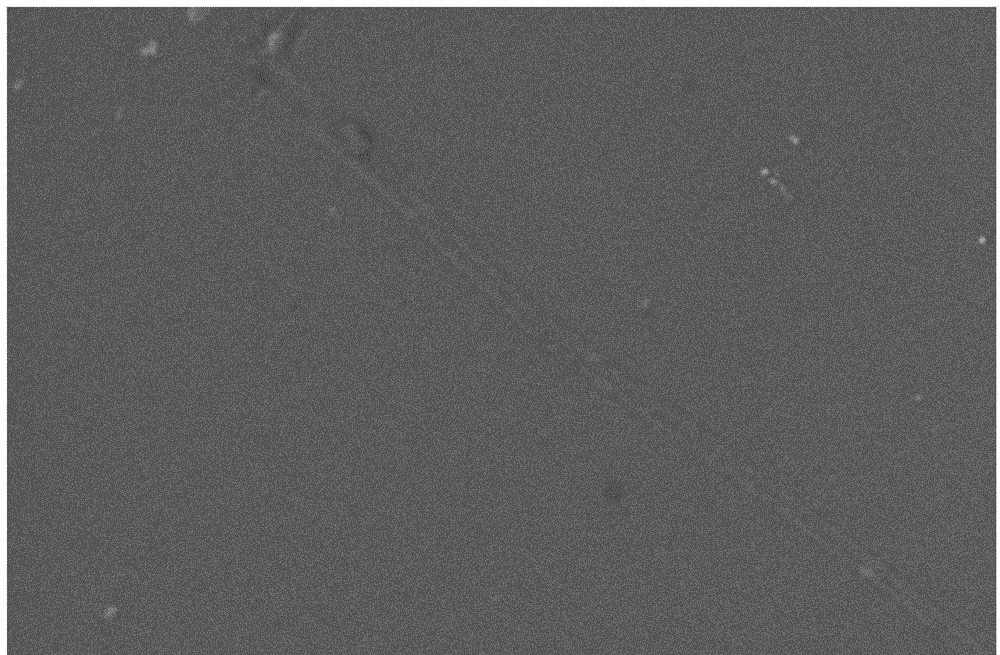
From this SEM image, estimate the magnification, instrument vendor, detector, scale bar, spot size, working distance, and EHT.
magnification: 10 K X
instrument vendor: FEI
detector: SE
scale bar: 2000 nm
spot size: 3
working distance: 5.7 mm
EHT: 12 kV